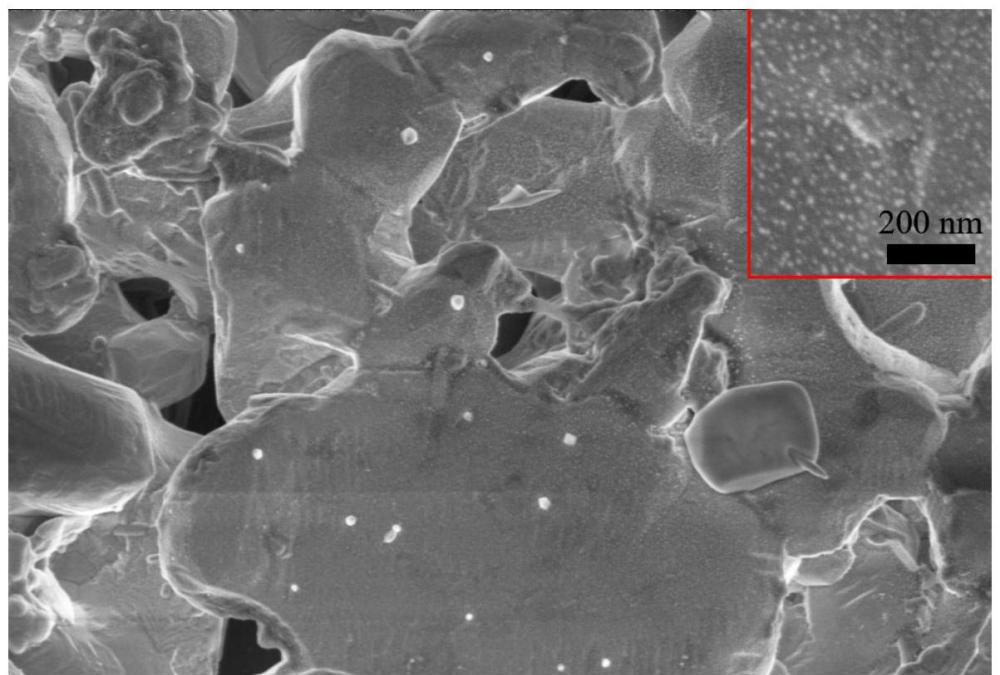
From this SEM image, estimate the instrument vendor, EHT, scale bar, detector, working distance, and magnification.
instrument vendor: Zeiss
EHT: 2 kV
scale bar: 1000 nm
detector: InLens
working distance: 6.2 mm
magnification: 10 K X